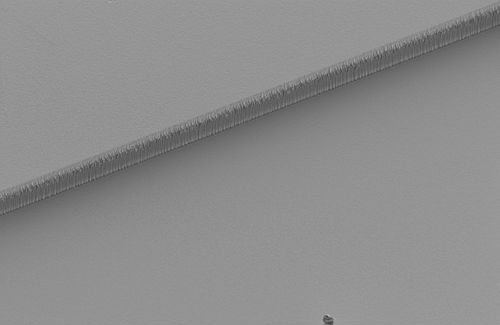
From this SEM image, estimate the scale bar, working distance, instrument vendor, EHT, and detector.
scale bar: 10000 nm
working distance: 12.8 mm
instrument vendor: Zeiss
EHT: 2.5 kV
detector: SE2